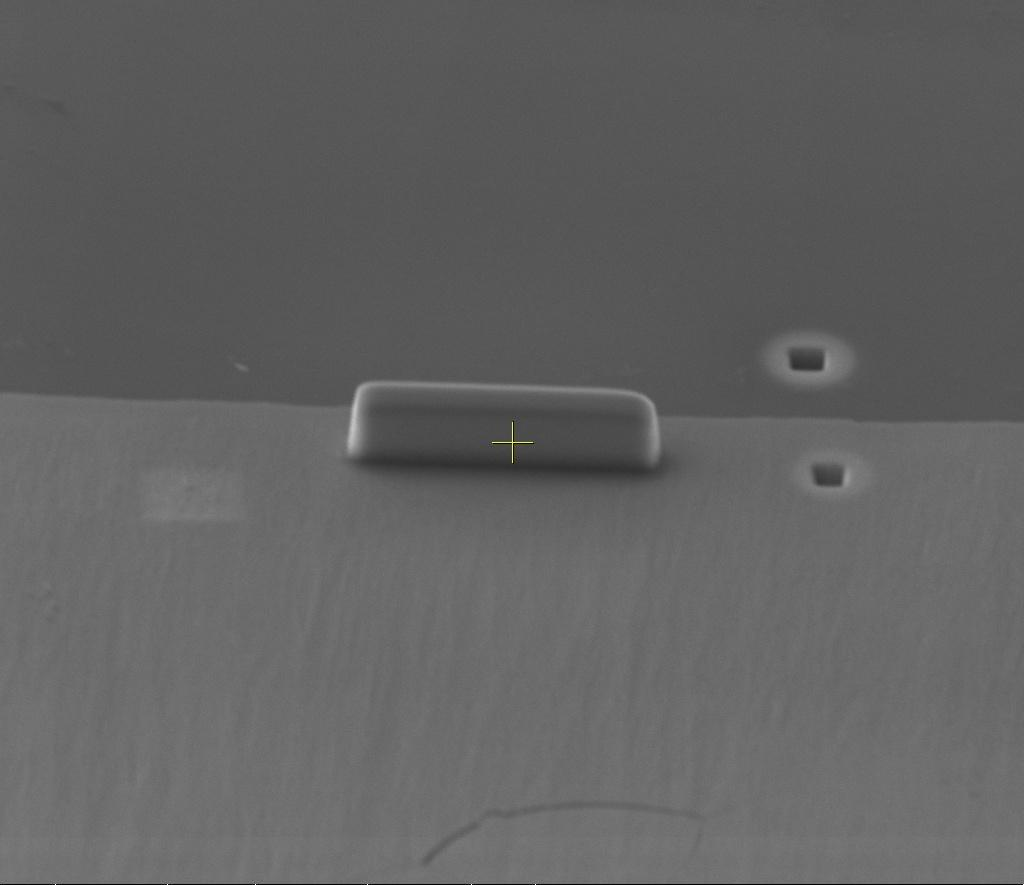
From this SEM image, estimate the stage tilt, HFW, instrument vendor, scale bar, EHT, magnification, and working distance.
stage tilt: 52°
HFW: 48.3 µm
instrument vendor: FEI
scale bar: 20000 nm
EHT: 10 kV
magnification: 3.088 K X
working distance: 15.1 mm